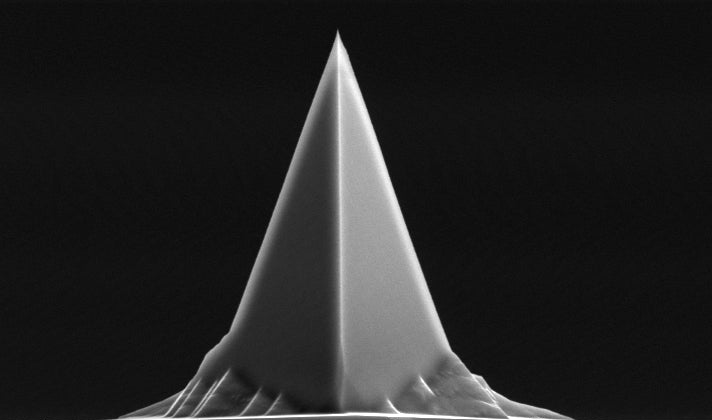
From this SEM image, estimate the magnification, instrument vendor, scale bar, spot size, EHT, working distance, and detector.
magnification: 5 K X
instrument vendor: FEI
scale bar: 5000 nm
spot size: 3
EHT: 10 kV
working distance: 22.5 mm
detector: SE